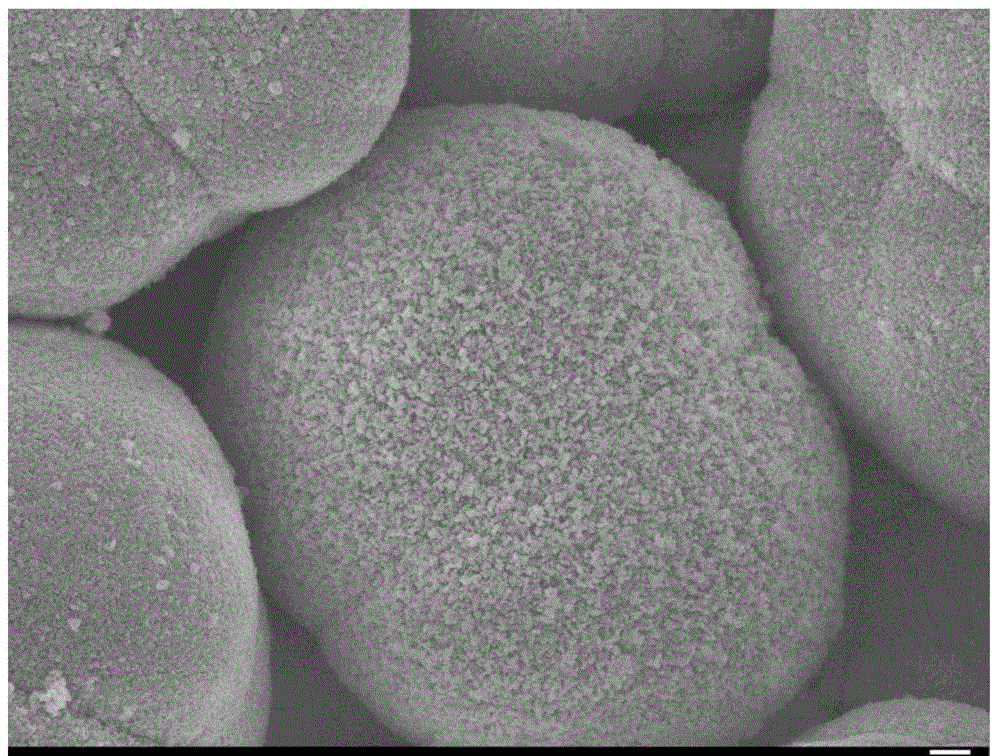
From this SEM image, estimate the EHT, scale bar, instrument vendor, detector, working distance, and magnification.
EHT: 10 kV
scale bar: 1000 nm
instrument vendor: JEOL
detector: SEI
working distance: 7.7 mm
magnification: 5 K X